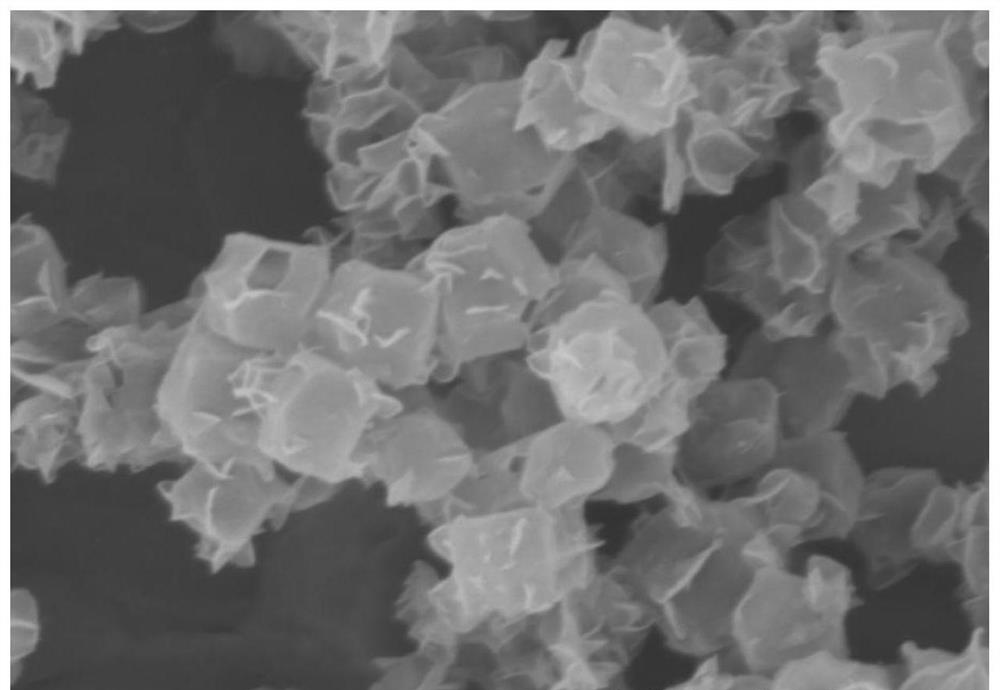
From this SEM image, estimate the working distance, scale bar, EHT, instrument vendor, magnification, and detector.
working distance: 10.2 mm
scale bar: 1000 nm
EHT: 3 kV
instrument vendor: Hitachi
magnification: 45 K X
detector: SE(M)